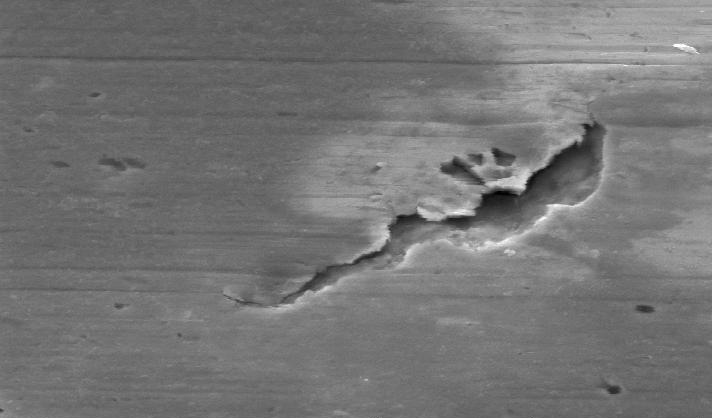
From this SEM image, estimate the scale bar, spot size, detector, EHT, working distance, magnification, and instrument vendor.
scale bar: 10000 nm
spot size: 3.5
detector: SE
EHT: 20 kV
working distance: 17 mm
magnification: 2 K X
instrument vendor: FEI